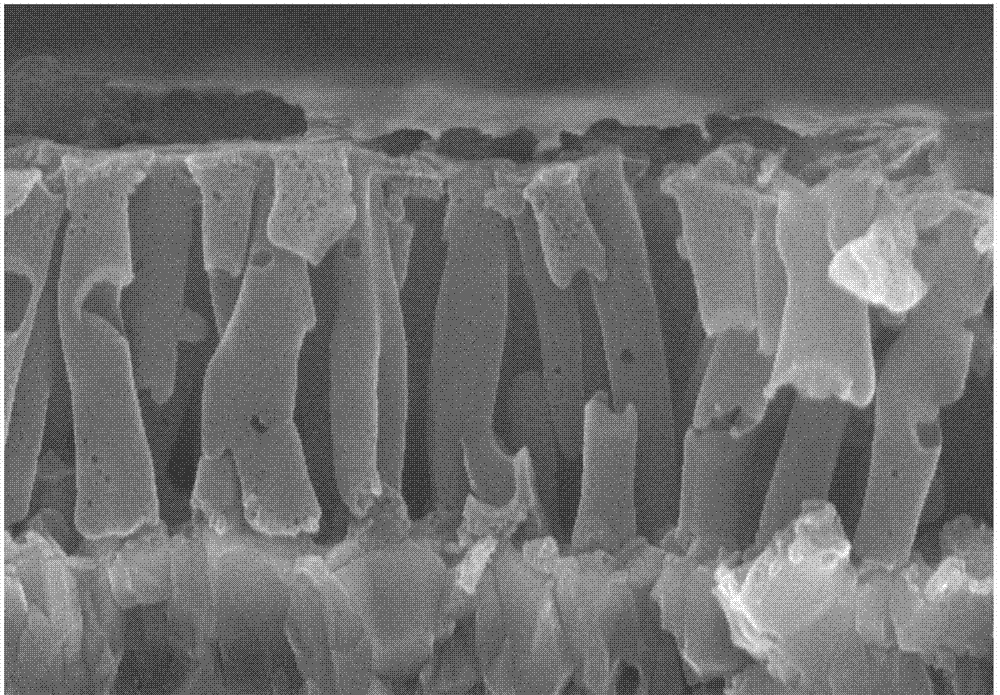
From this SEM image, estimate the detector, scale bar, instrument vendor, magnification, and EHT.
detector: SE(UL)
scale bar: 1000 nm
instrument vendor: Hitachi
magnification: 50 K X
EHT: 10 kV